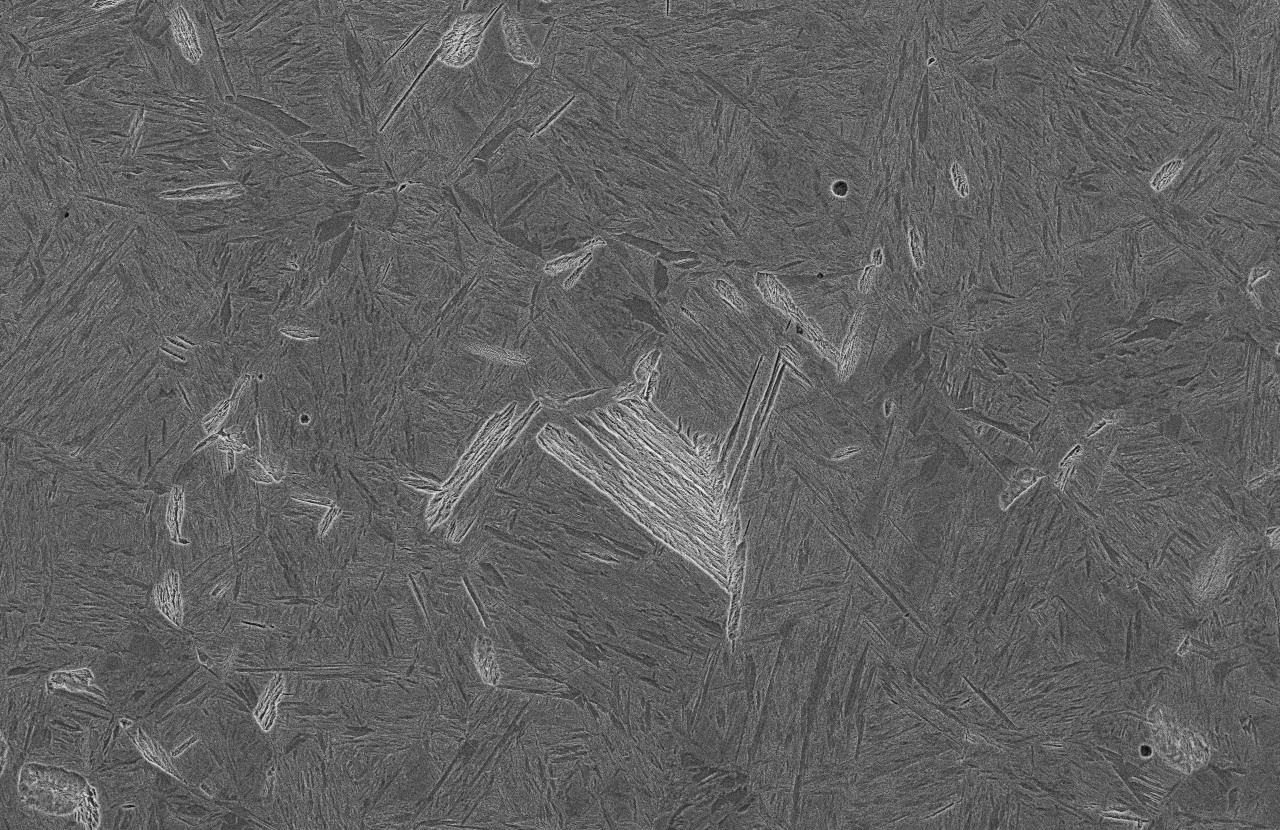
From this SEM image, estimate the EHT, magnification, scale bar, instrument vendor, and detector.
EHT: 15 kV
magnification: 0.5 K X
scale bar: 50000 nm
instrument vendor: JEOL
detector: SEI